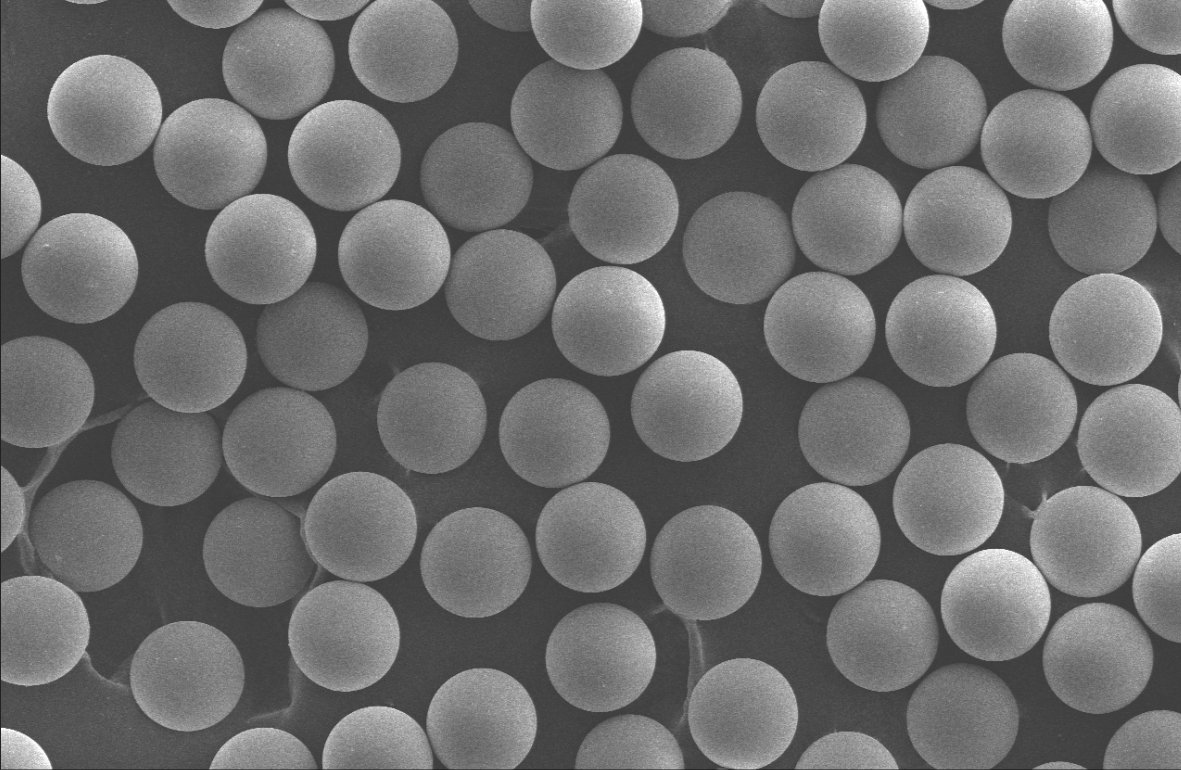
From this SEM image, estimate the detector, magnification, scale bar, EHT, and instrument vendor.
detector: SEI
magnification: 0.1 K X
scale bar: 100000 nm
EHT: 20 kV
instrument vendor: JEOL